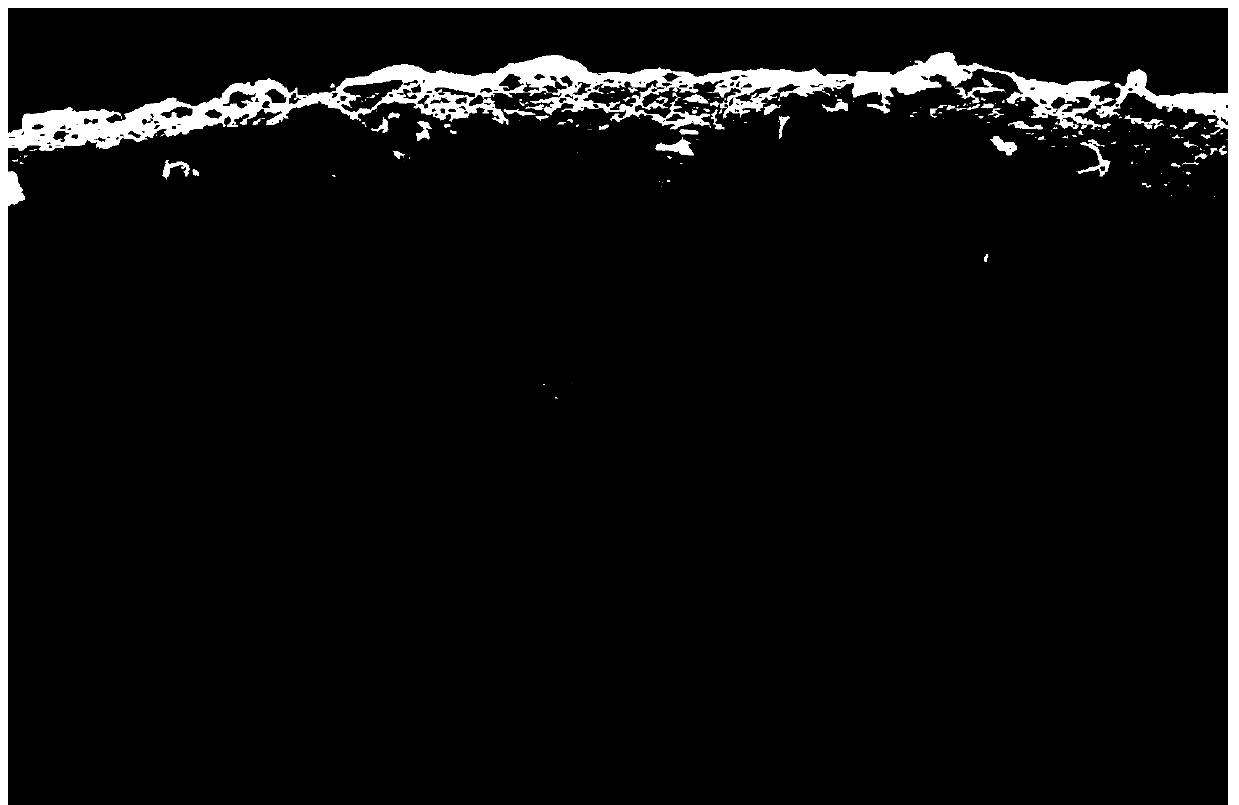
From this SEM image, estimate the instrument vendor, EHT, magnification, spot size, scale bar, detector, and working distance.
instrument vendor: FEI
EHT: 15 kV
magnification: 0.2 K X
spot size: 3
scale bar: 100000 nm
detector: SE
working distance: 6 mm